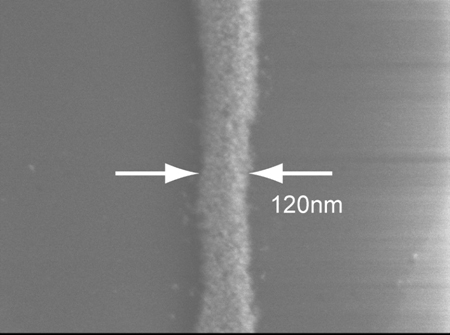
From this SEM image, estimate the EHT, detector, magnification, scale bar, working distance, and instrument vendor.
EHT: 5 kV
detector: SEI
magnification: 70 K X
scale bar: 100 nm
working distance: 14 mm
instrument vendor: JEOL